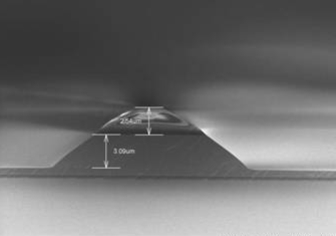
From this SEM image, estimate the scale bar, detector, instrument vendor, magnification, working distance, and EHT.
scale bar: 10000 nm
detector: SE(M)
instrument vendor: Hitachi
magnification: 4.02 K X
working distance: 3.7 mm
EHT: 5 kV